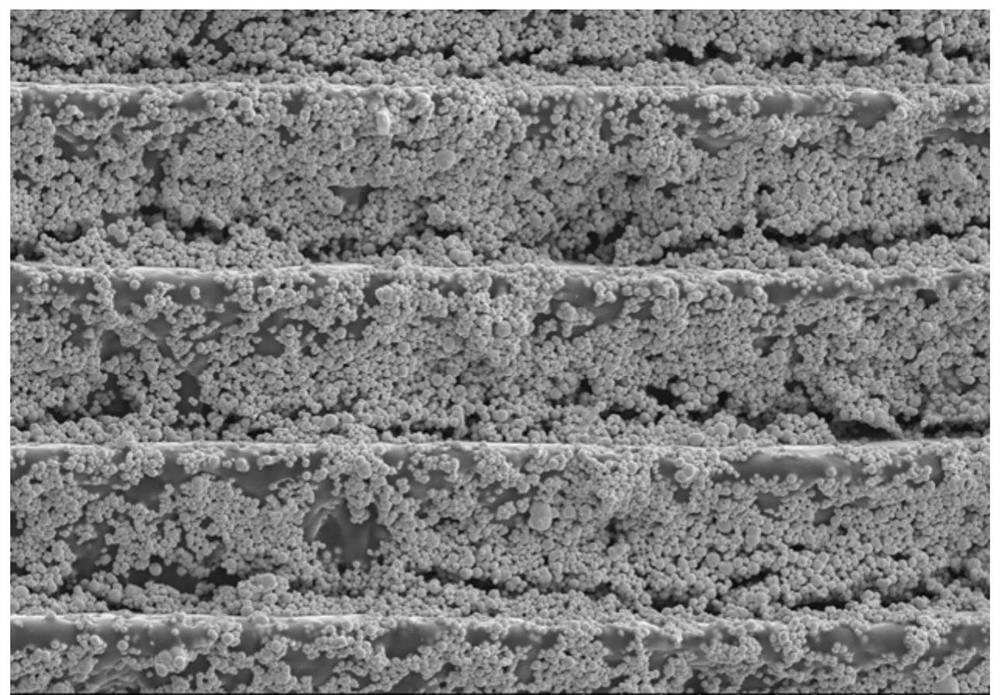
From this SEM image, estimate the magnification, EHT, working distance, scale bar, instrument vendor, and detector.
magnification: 0.5 K X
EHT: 15 kV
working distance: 31 mm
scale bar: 100000 nm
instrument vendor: Hitachi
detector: SE